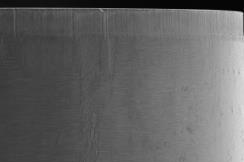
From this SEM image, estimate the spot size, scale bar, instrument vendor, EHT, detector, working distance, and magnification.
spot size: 85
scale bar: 500000 nm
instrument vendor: JEOL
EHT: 20 kV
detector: SEI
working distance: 32 mm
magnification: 0.045 K X